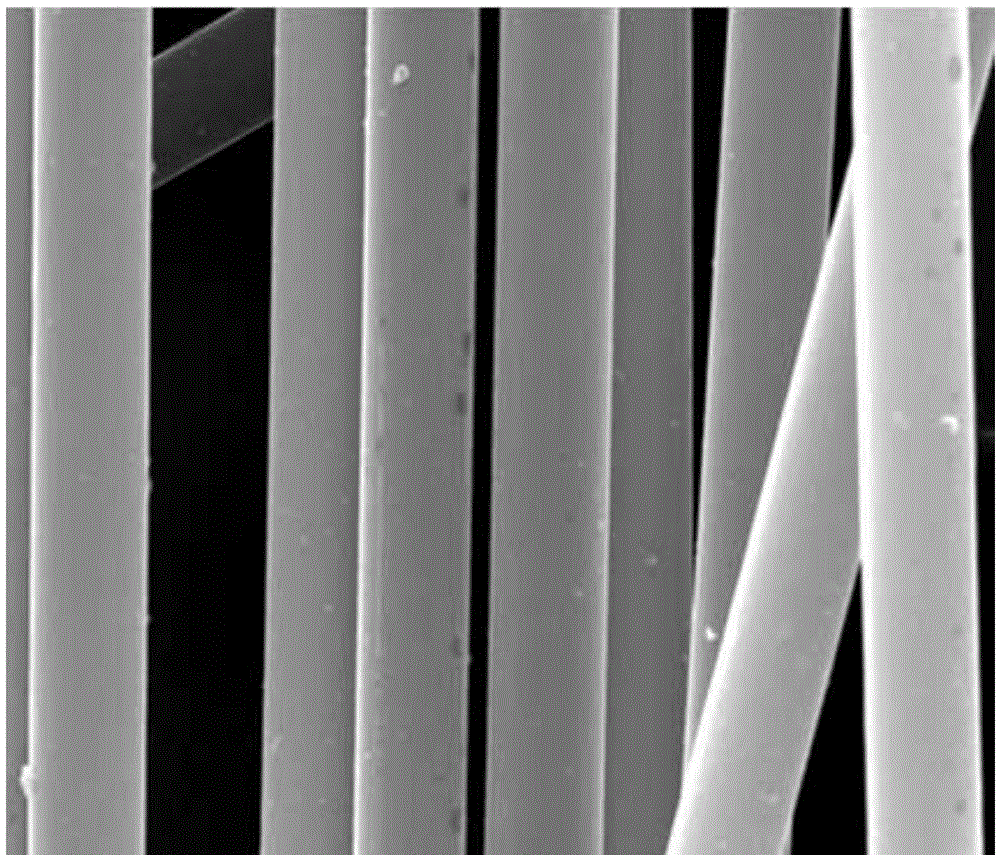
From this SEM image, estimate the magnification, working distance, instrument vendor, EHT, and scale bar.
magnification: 5 K X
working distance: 15.3 mm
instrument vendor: FEI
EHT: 30 kV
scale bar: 20000 nm